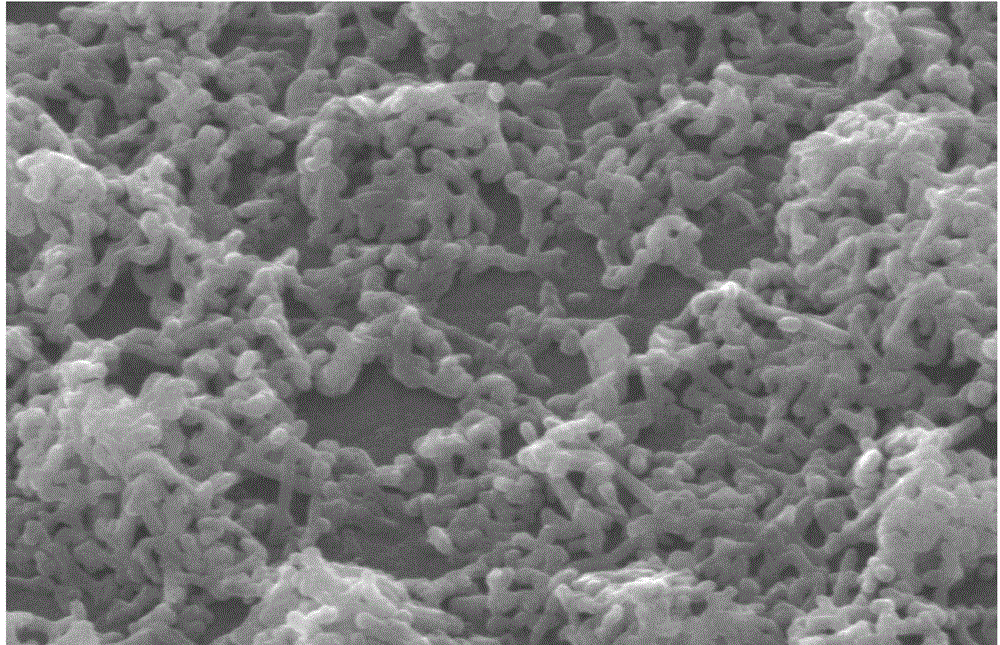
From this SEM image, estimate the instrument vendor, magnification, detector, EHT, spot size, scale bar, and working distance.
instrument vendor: FEI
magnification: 20.003 K X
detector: SE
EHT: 20 kV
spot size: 3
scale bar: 1000 nm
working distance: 6.5 mm